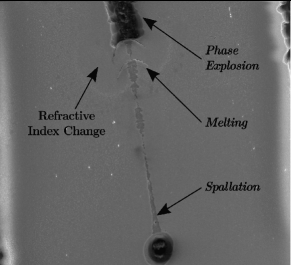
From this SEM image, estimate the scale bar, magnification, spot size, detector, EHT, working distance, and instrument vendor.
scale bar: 100000 nm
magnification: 1.2 K X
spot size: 4.5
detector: LFD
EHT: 16.7 kV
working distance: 9.3 mm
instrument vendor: FEI